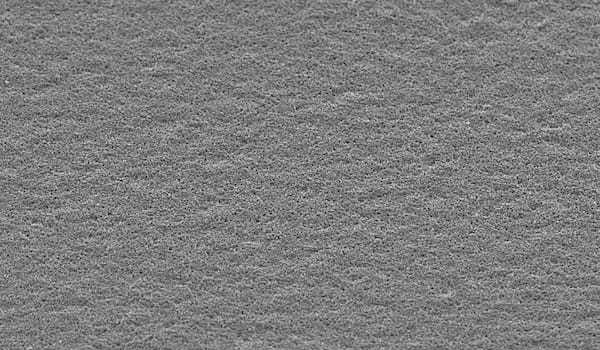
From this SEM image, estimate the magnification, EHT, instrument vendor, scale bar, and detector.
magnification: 0.2 K X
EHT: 20 kV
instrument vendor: JEOL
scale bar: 100000 nm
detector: SEI